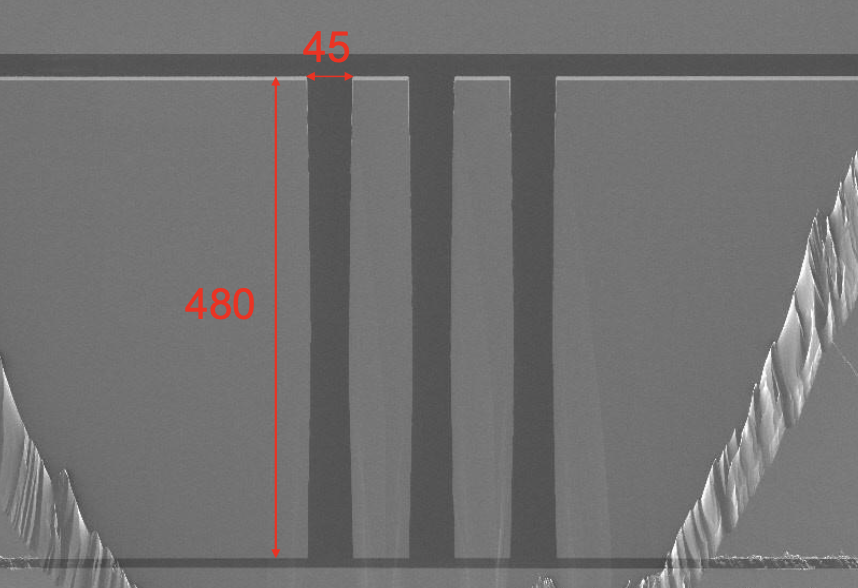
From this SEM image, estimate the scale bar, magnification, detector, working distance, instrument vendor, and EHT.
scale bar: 300000 nm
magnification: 0.15 K X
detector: LM(UL)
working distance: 8.3 mm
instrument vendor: Hitachi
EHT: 5 kV